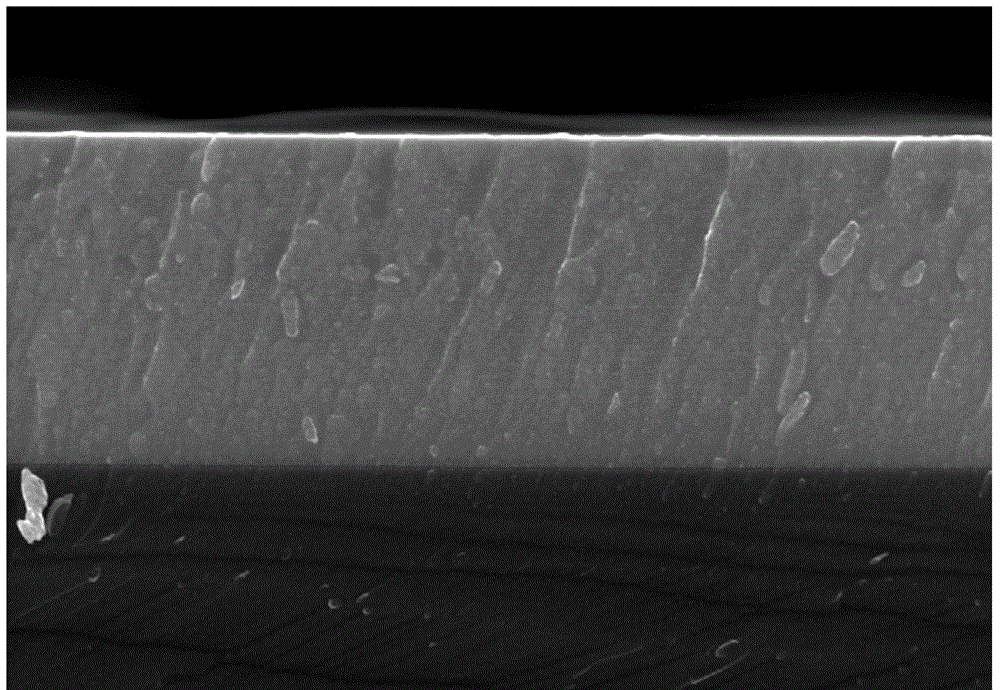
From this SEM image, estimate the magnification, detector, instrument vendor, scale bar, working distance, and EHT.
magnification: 10 K X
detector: SE(M)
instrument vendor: Hitachi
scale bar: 5000 nm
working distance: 4.8 mm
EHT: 20 kV